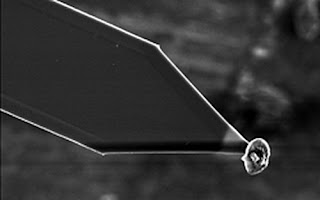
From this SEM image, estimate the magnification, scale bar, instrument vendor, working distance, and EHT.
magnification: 1.6 K X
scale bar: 10000 nm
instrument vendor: JEOL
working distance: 11 mm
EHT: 10 kV